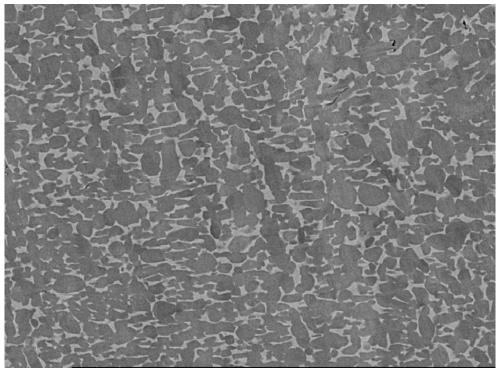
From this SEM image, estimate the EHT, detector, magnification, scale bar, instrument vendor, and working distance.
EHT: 15 kV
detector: COMP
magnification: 1 K X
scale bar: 10000 nm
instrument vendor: JEOL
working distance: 10 mm